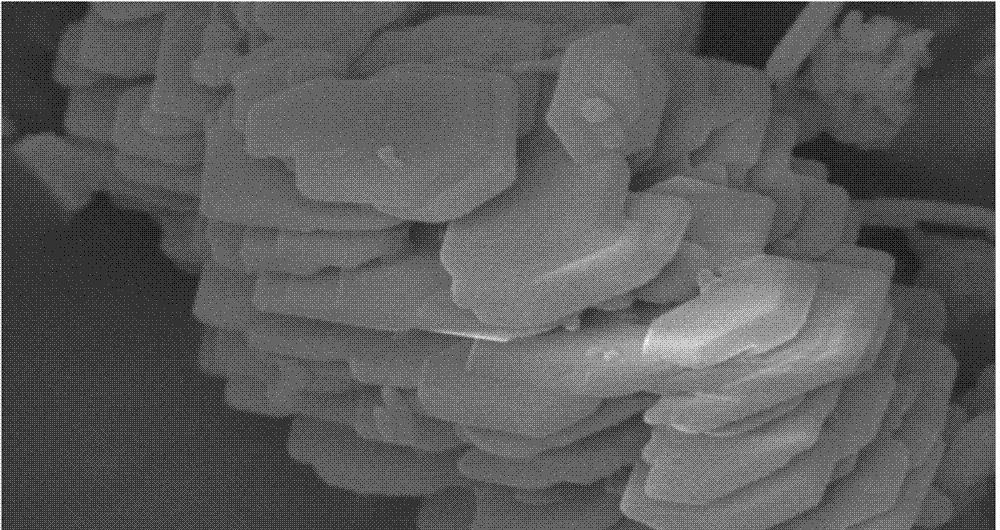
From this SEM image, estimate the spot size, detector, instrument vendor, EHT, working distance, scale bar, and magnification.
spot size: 4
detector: SE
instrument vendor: FEI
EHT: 20 kV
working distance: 7 mm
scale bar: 5000 nm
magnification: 12.8 K X